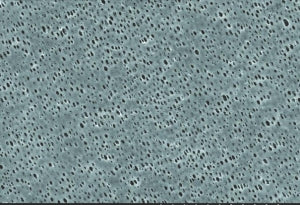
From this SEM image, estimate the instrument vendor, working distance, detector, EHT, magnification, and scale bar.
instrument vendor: Hitachi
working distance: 32.7 mm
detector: SE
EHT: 15 kV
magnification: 1 K X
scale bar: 30000 nm